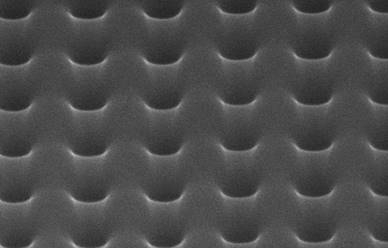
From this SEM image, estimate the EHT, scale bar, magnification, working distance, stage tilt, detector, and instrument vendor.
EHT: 30 kV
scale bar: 300 nm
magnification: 200 K X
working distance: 4 mm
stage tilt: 52°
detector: ETD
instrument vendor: FEI